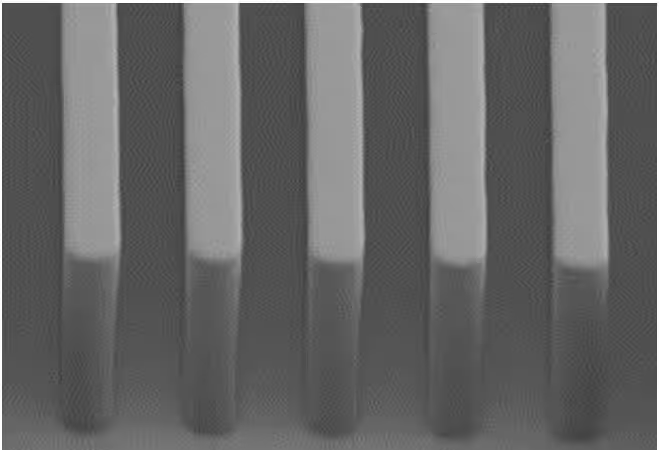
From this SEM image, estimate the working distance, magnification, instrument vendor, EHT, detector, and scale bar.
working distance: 24.9 mm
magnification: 8 K X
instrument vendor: Hitachi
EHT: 2 kV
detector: SE(UL)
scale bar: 5000 nm